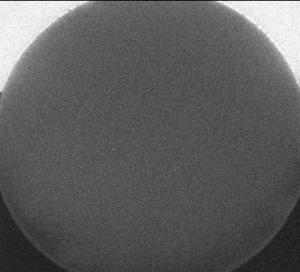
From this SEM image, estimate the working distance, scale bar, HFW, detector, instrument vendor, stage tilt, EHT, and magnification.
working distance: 4.3 mm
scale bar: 500 nm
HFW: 1.6 µm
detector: TLD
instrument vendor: FEI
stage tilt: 52°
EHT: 10 kV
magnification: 79.985 K X